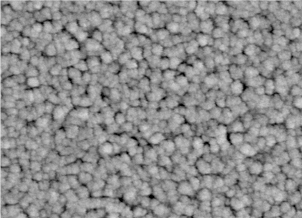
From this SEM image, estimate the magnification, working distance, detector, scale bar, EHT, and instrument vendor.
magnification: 100 K X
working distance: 9.5 mm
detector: SEI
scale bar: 100 nm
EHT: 5 kV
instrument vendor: JEOL